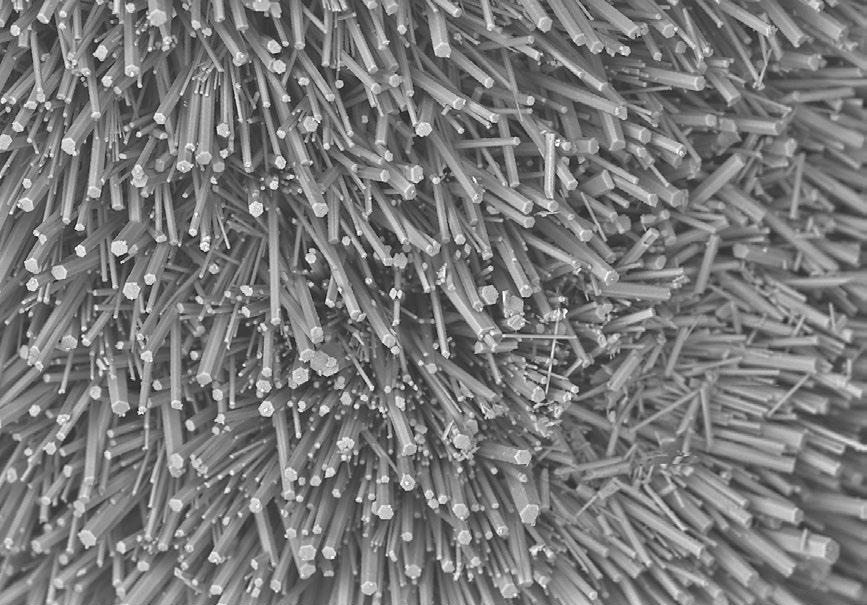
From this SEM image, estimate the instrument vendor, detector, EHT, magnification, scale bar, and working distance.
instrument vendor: Hitachi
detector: BSE3D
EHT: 15 kV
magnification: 0.27 K X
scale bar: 200000 nm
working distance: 12.5 mm